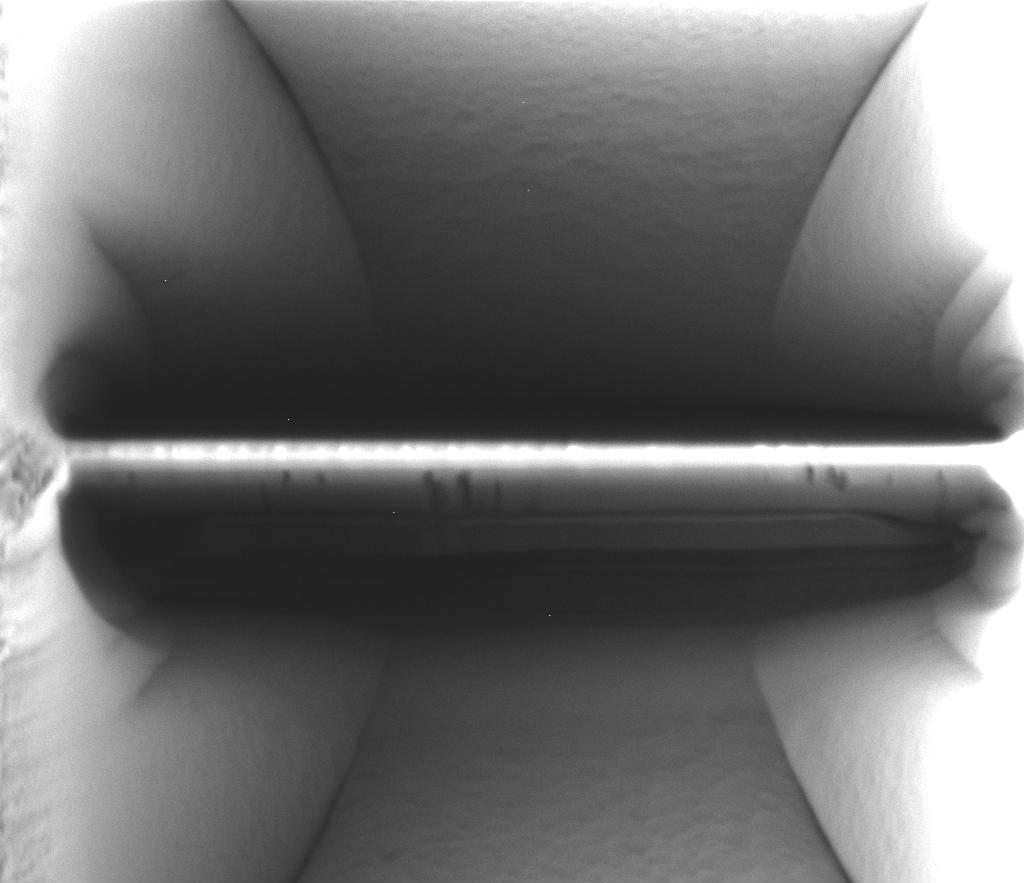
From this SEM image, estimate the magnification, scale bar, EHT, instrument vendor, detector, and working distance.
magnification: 6.5 K X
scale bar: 5000 nm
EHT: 30 kV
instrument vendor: FEI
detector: CDEM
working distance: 19 mm